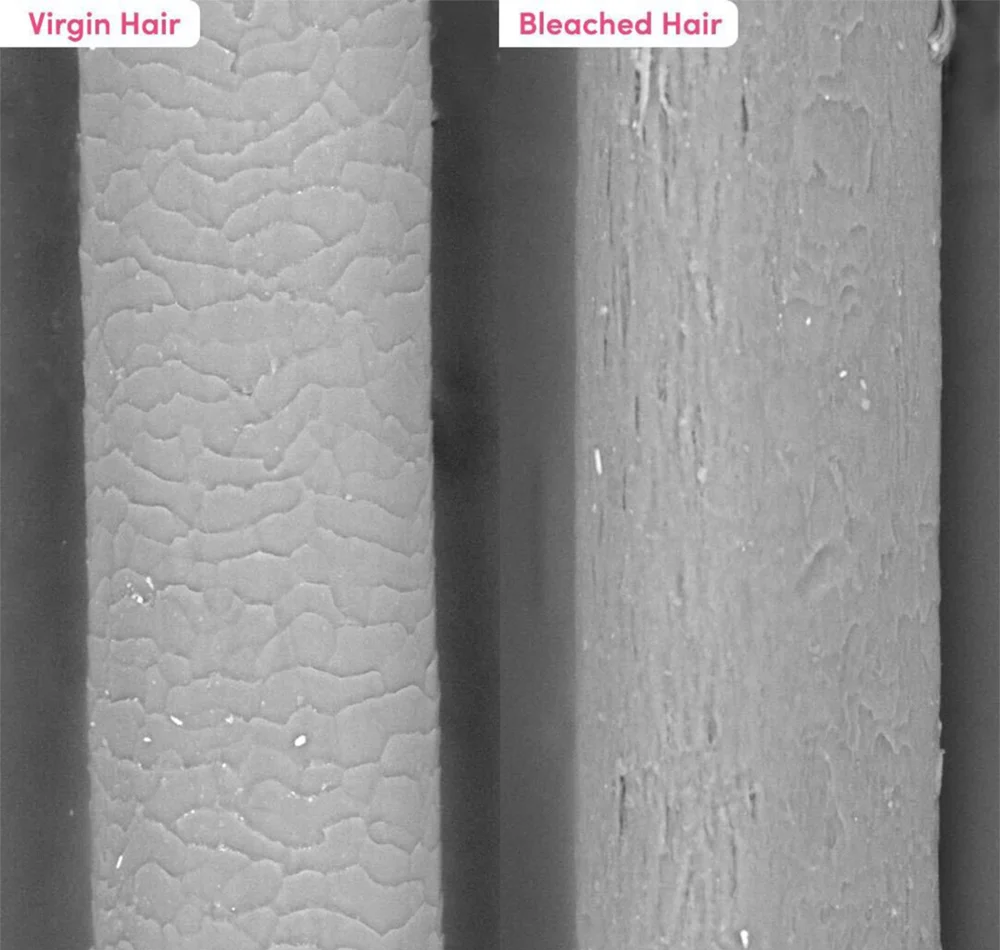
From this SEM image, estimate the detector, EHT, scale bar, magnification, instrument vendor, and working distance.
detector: BSE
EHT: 15 kV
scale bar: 100000 nm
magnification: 0.4 K X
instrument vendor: Hitachi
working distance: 8 mm